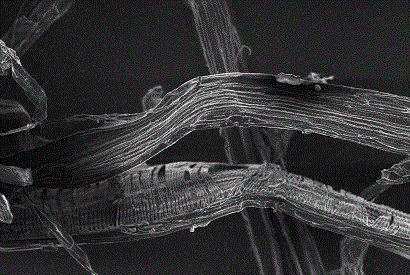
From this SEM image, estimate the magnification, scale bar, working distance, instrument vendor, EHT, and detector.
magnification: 0.5 K X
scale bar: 20000 nm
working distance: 3.7 mm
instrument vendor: Zeiss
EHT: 5 kV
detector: InLens